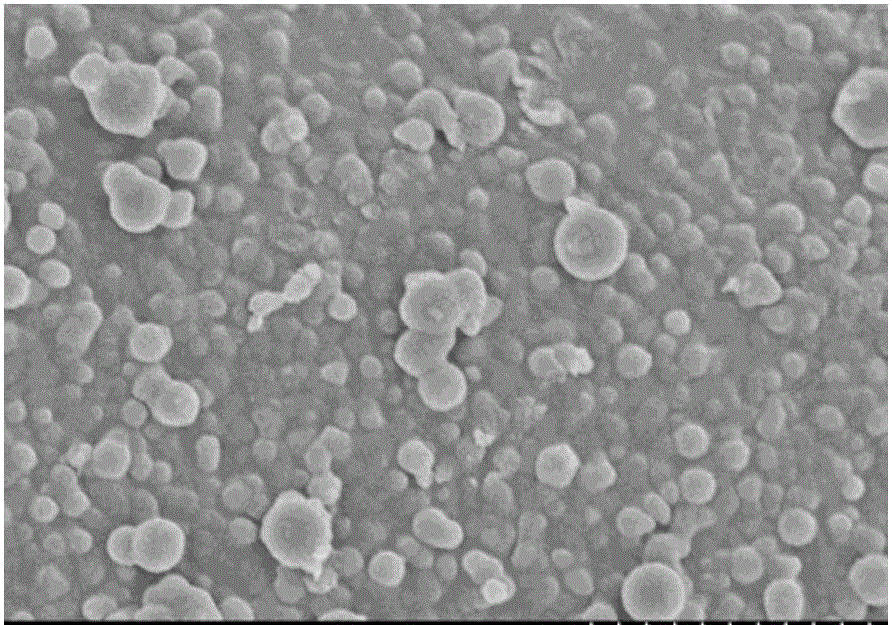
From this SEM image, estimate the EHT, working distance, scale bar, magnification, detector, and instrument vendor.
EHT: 3 kV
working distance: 9.4 mm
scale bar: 2000 nm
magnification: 20 K X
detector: SE(M)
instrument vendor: Hitachi